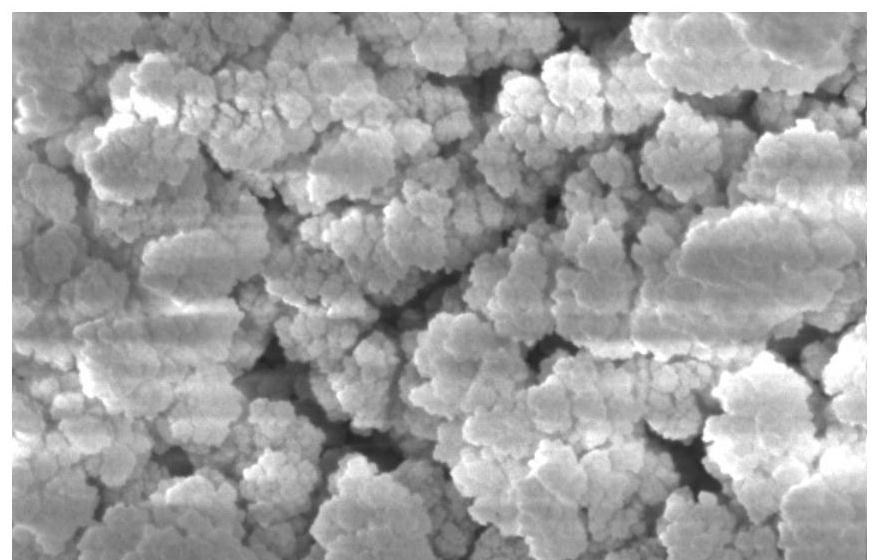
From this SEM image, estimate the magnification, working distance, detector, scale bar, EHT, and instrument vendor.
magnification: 5 K X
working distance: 10.7 mm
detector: SE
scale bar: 5000 nm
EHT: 10 kV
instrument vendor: FEI